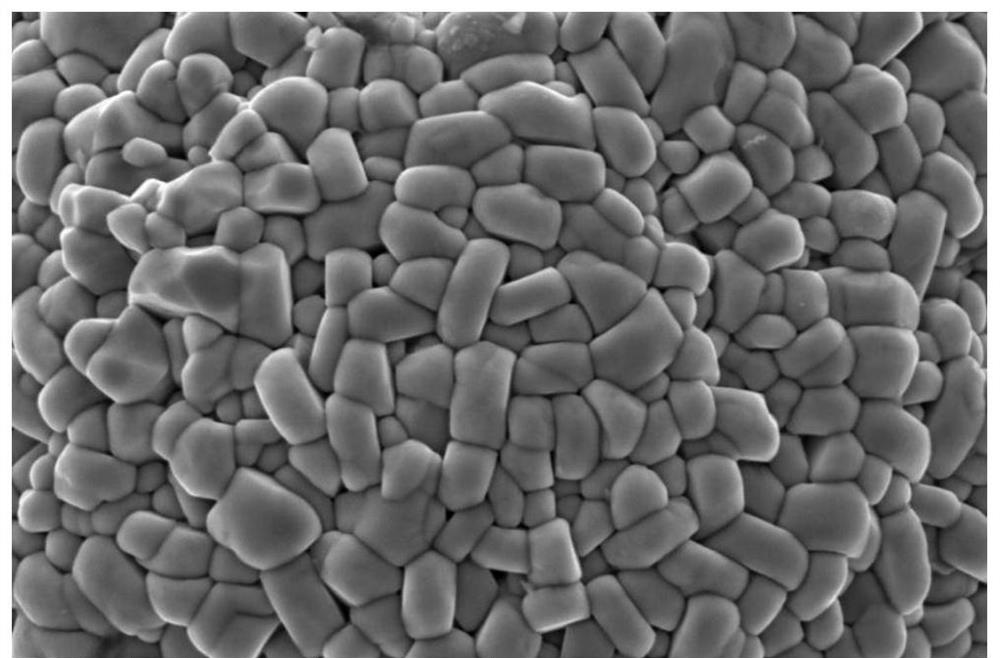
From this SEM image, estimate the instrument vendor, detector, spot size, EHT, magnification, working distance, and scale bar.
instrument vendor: FEI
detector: ETD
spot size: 3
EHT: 5 kV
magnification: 50 K X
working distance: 10.3 mm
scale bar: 3000 nm